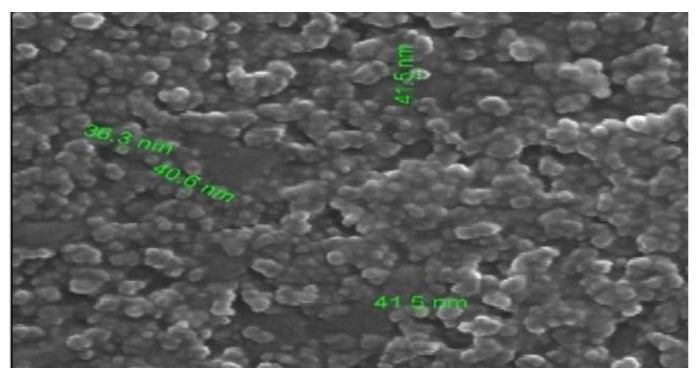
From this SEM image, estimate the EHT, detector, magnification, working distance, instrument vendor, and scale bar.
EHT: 30 kV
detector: ETD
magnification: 60 K X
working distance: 9.6 mm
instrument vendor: FEI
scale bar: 500 nm